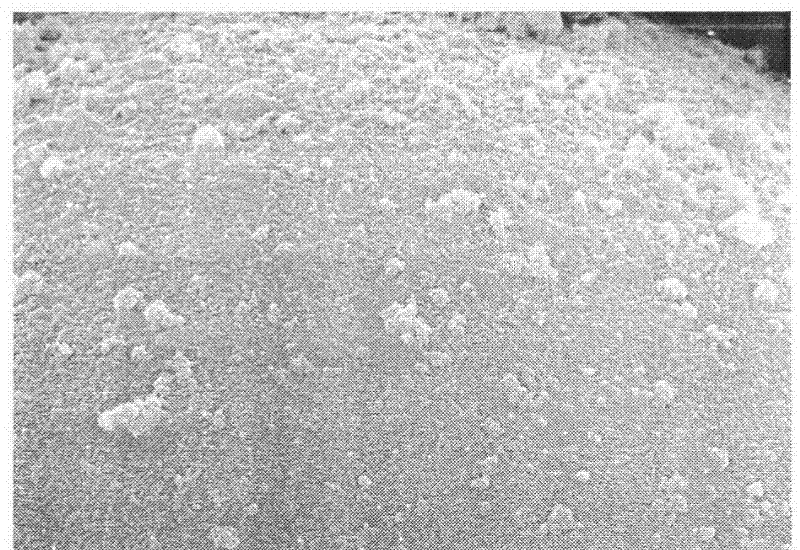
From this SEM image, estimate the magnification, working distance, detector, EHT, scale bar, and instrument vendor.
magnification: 15 K X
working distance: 15.2 mm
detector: SE(M)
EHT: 15 kV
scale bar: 3000 nm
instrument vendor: Hitachi